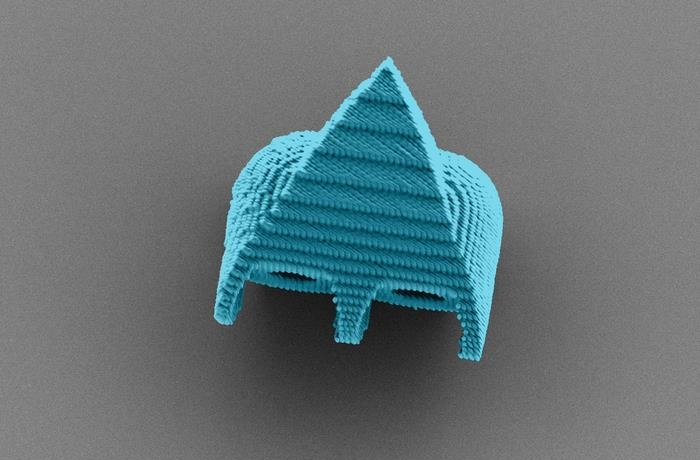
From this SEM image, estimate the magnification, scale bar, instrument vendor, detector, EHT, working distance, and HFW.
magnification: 1.02 K X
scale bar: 10000 nm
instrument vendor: Zeiss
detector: SE2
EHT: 1.5 kV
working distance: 4 mm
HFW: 112.3 µm